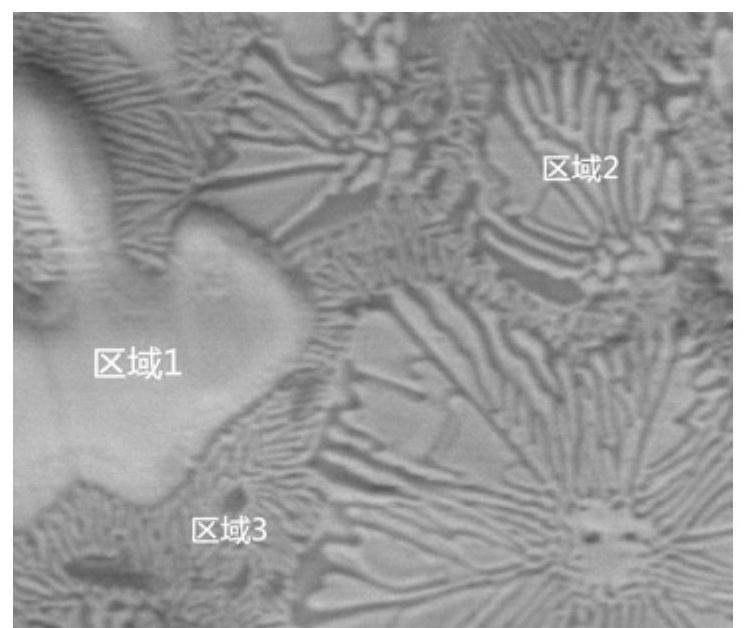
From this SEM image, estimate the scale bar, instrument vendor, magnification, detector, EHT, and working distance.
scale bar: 20000 nm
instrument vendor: FEI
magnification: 3 K X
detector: BSED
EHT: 20 kV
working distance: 14.8 mm